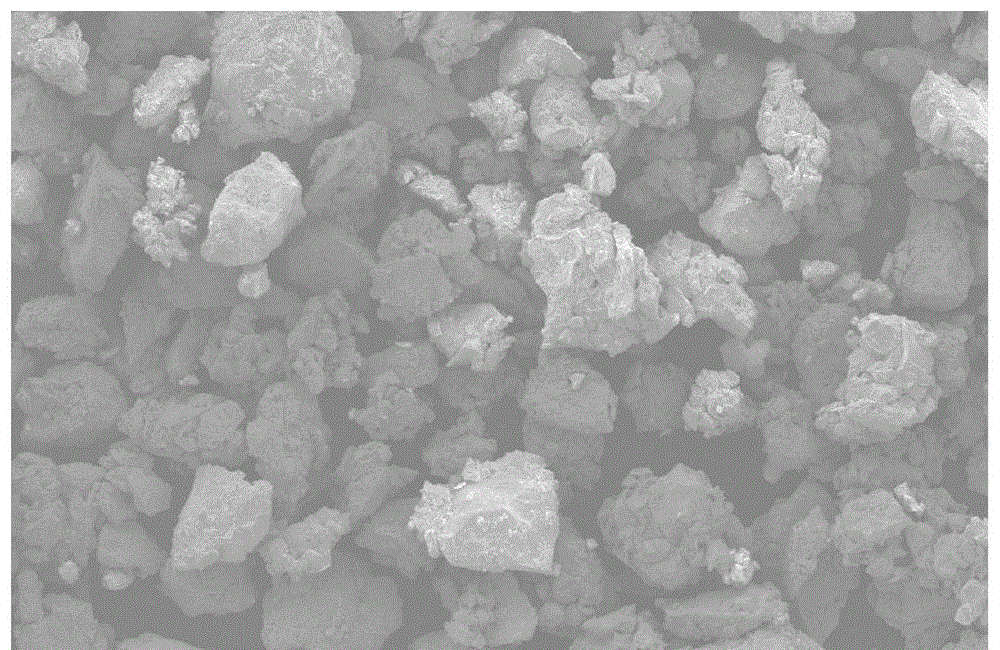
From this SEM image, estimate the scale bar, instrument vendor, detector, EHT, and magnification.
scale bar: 500000 nm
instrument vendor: JEOL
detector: SEI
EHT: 20 kV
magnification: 0.05 K X